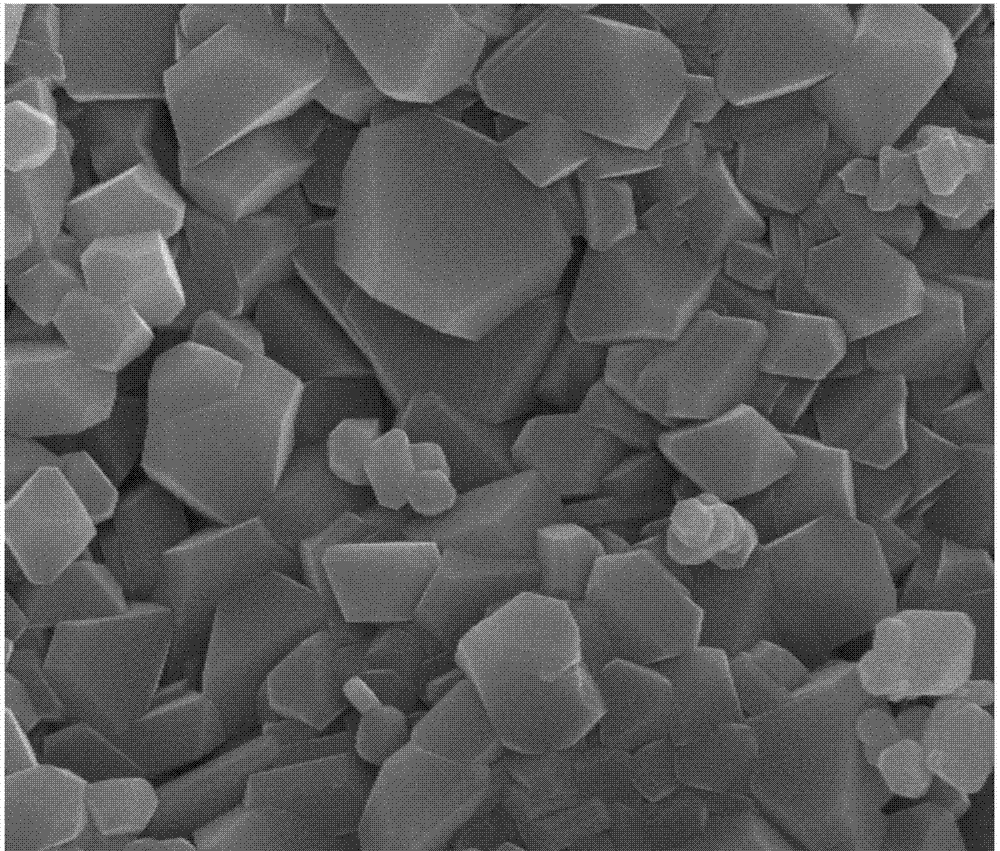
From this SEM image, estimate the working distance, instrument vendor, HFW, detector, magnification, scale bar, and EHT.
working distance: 5.2 mm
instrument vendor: FEI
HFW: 8 µm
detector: TLD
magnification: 30 K X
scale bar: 2000 nm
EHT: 18 kV